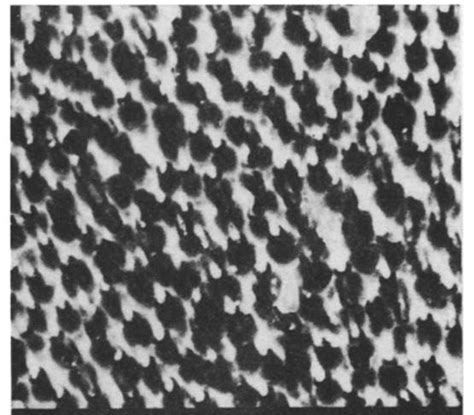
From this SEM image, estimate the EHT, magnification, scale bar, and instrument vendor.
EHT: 15 kV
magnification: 6.5 K X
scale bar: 1000 nm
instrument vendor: JEOL